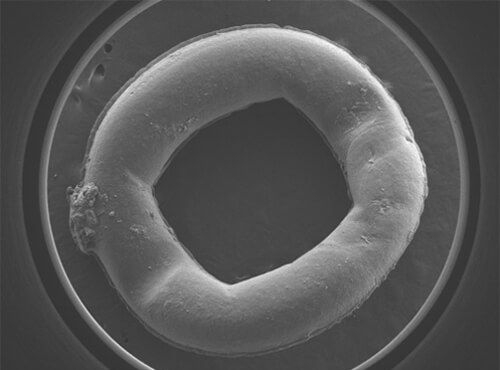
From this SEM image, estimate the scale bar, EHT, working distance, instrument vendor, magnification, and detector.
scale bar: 1000000 nm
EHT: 15 kV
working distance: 8 mm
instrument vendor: JEOL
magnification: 0.025 K X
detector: LEI